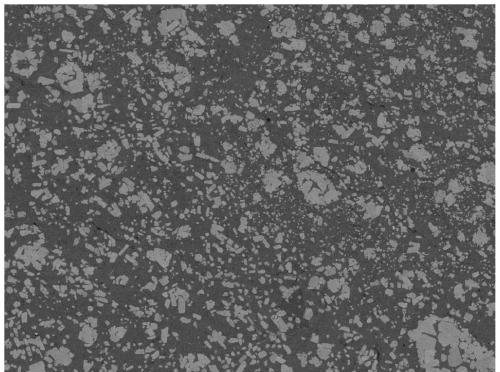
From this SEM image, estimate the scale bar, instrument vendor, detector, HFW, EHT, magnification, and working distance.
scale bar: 50000 nm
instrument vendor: Tescan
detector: BSE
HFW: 277 µm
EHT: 10 kV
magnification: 1 K X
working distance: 14.85 mm